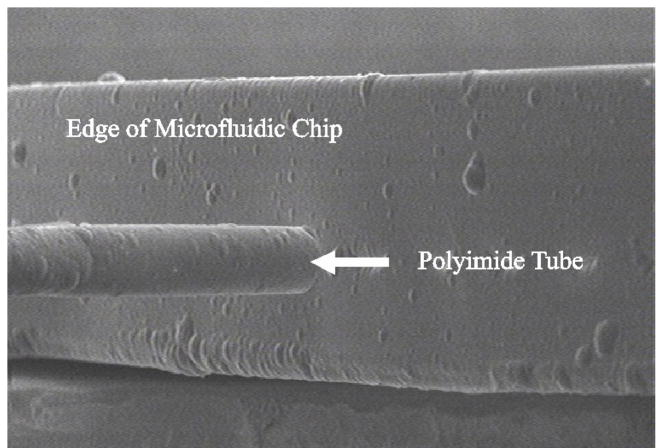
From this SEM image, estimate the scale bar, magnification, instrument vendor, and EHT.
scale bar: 1000000 nm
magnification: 0.42 K X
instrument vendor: other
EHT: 30 kV